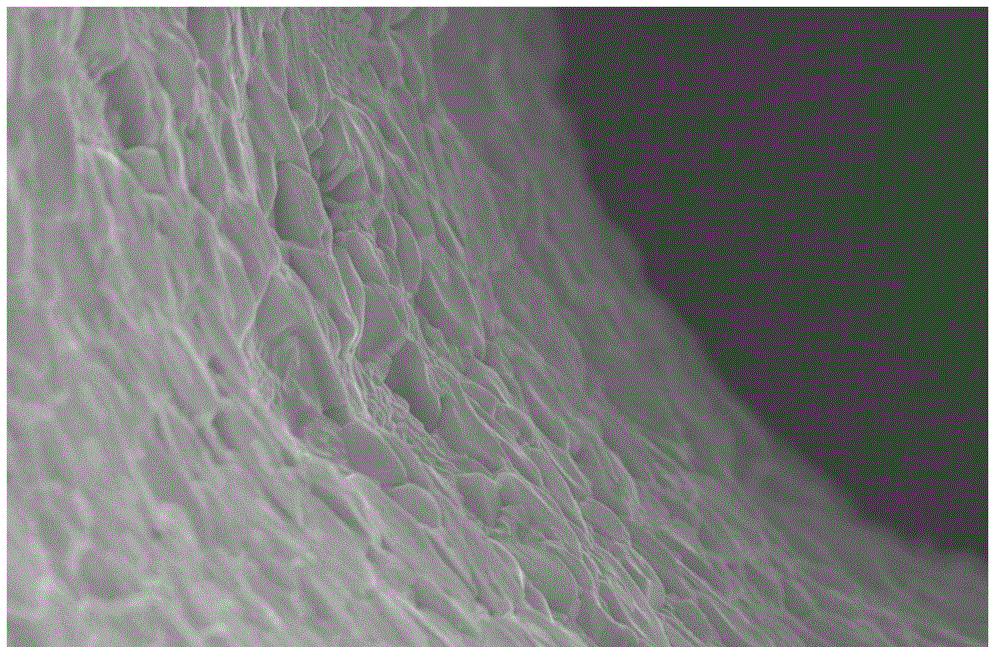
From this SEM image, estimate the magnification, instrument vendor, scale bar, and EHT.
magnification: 4 K X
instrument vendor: JEOL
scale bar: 5000 nm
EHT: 20 kV